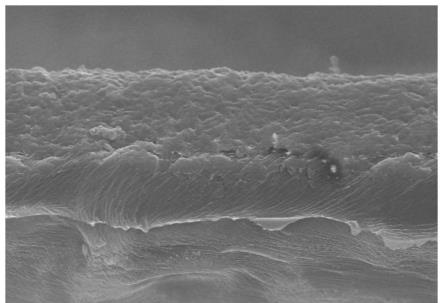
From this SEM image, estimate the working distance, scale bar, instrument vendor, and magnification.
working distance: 13.4 mm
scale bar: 20000 nm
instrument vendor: Hitachi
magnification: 2.5 K X